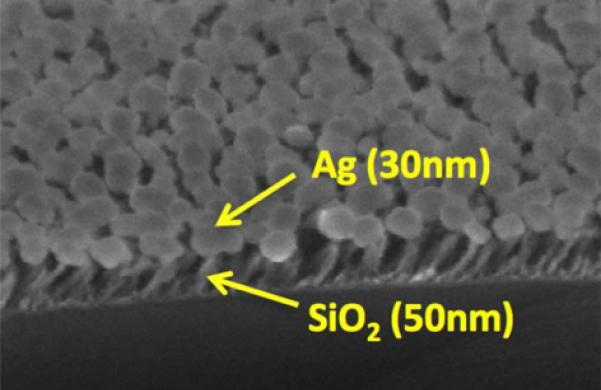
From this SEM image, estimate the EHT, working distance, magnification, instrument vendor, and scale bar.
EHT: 15 kV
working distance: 9.2 mm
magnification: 200 K X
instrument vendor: Hitachi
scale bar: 200 nm